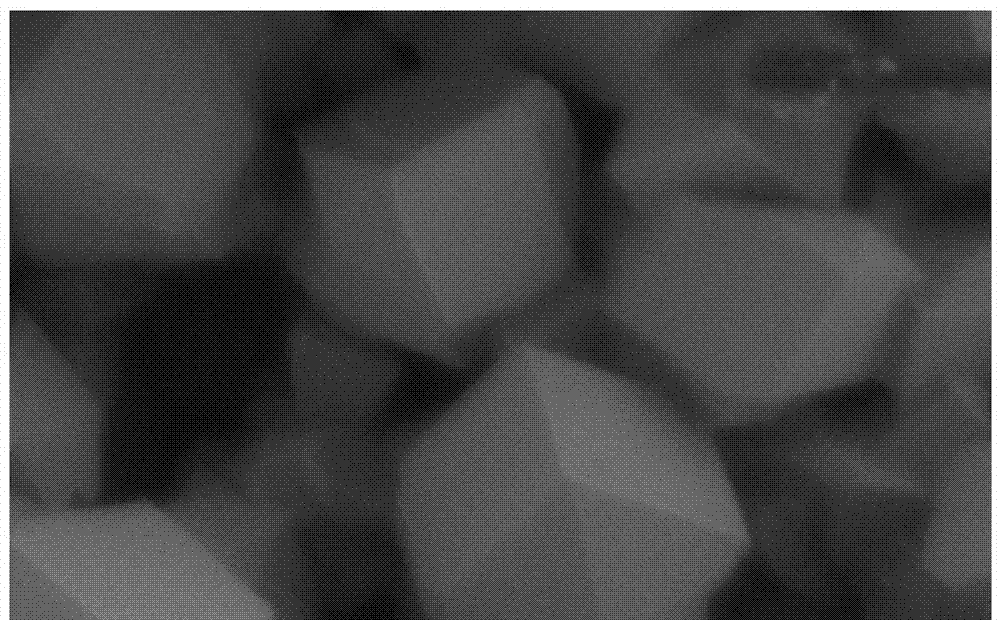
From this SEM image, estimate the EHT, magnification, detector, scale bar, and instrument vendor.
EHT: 15 kV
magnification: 1 K X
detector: SEI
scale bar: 20000 nm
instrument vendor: JEOL